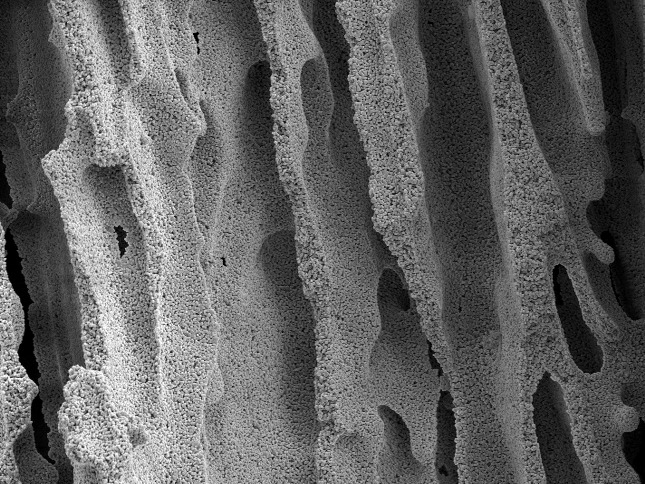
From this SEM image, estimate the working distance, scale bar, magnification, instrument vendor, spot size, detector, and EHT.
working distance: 7.2 mm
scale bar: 10000 nm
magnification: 2.147 K X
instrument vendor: FEI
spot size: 3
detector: SE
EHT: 5 kV